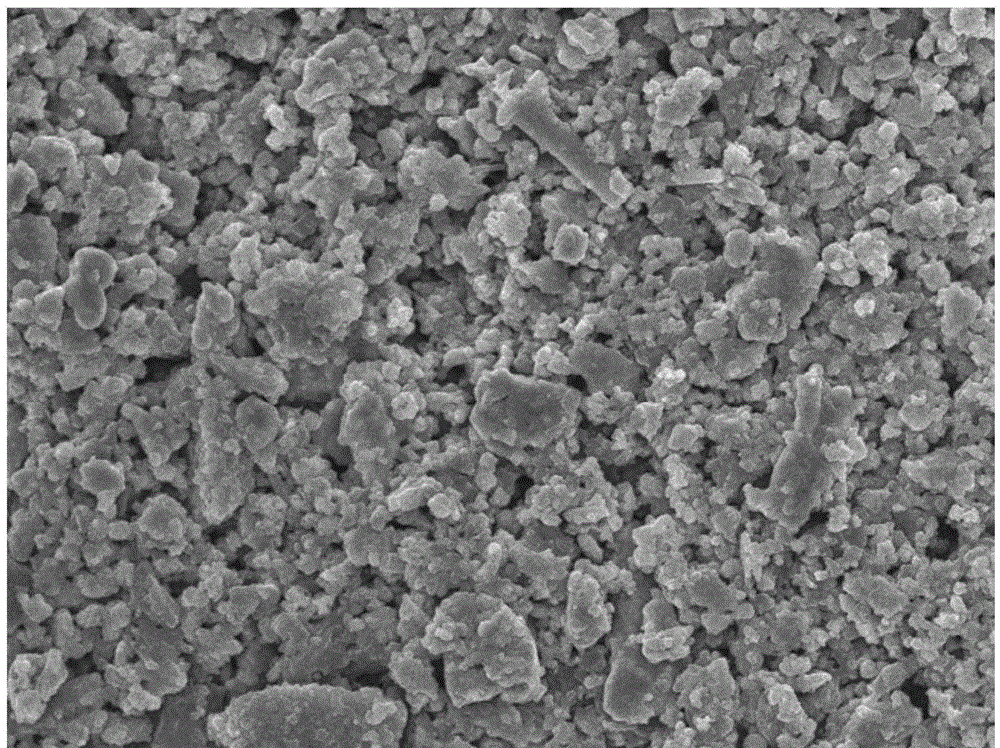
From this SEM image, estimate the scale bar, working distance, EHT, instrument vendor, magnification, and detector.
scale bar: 1000 nm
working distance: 8 mm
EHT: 10 kV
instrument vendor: JEOL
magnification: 5 K X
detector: SEI